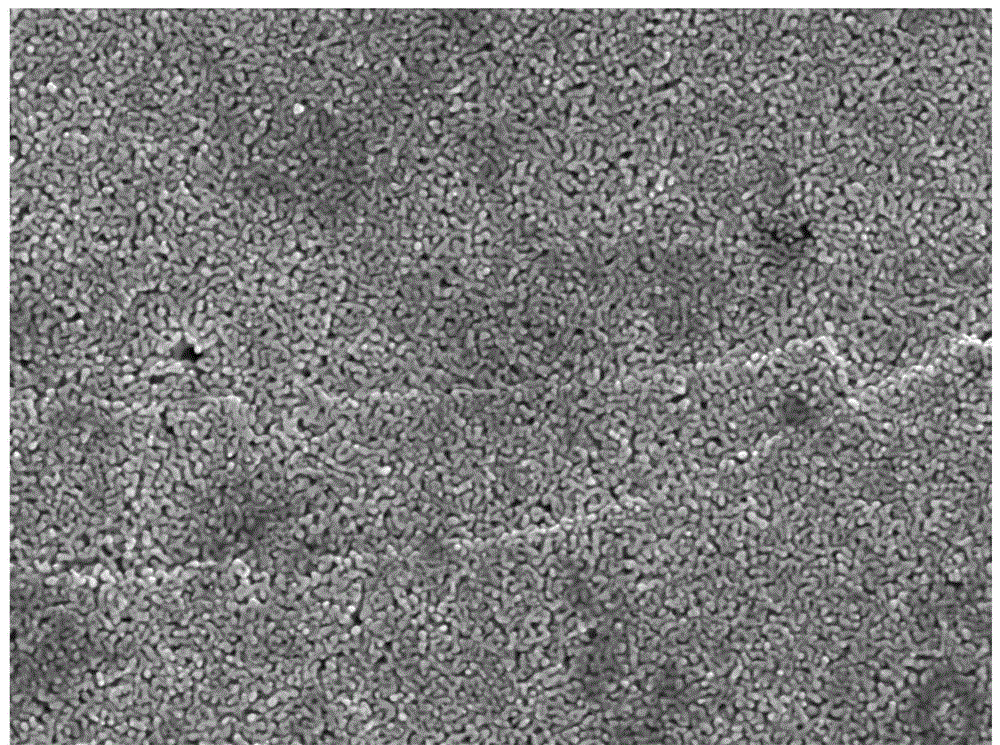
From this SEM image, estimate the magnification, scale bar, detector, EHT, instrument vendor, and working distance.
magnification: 40 K X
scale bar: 100 nm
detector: SEI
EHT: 5 kV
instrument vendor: JEOL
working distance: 8.4 mm